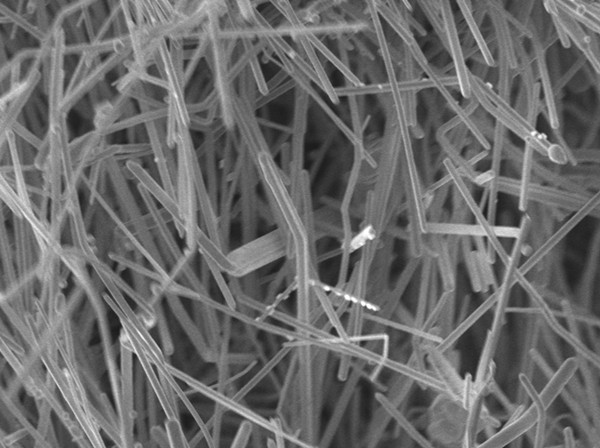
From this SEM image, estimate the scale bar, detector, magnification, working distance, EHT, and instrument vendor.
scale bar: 1000 nm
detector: UED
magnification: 10 K X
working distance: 3 mm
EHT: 3 kV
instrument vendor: JEOL